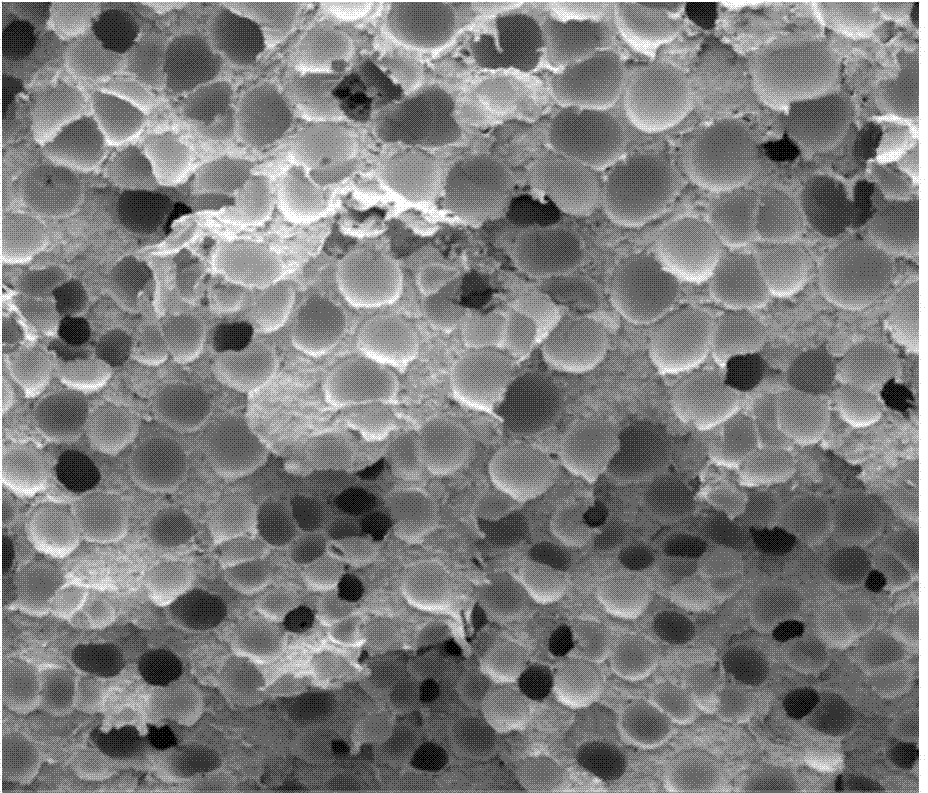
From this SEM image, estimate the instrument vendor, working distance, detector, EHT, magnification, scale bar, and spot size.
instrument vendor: FEI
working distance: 8.9 mm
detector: ETD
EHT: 2 kV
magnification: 0.2 K X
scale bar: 500000 nm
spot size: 2.5